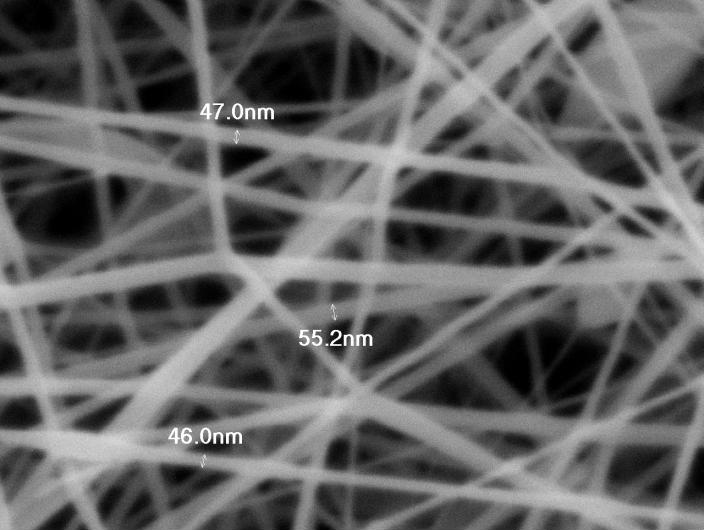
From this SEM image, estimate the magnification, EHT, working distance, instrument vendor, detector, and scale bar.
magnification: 50 K X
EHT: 5 kV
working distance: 10 mm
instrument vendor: JEOL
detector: SEI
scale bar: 100 nm